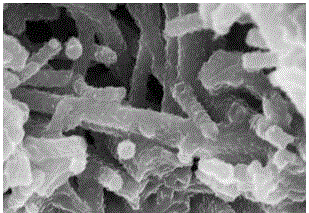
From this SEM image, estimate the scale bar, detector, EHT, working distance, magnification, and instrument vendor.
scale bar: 1000 nm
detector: SE(M)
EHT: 5 kV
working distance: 7.1 mm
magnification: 50 K X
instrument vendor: Hitachi